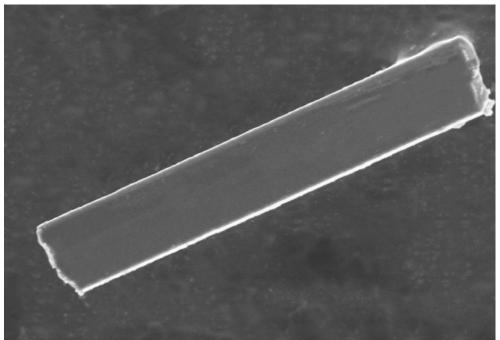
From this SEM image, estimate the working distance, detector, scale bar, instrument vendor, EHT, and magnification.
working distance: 8.2 mm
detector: SE(UL)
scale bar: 10000 nm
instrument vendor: Hitachi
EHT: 5 kV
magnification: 3.5 K X